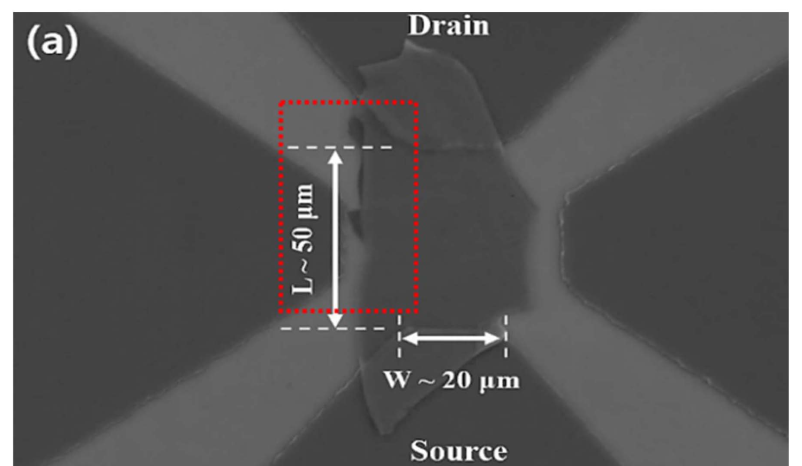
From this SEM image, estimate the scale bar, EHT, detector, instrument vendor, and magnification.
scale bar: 20000 nm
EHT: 20 kV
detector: SE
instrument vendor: Hitachi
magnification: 0.7 K X